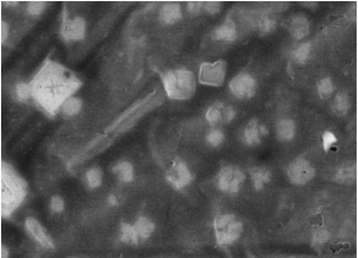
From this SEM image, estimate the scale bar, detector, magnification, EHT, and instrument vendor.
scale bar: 10000 nm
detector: BSE Detector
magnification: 10 K X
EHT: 30 kV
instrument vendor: Tescan